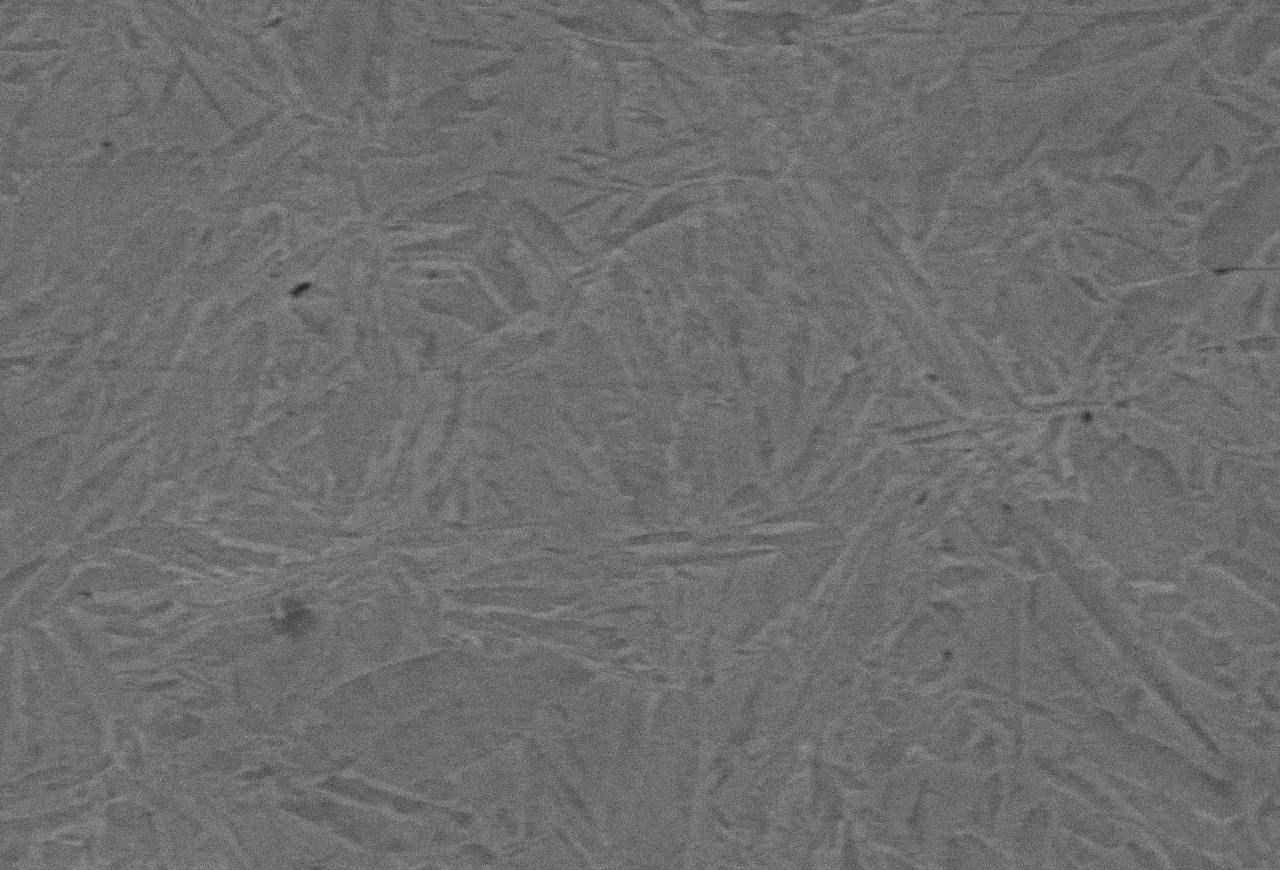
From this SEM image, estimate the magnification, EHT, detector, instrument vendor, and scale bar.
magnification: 4 K X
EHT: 15 kV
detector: SEI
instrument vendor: JEOL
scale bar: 5000 nm